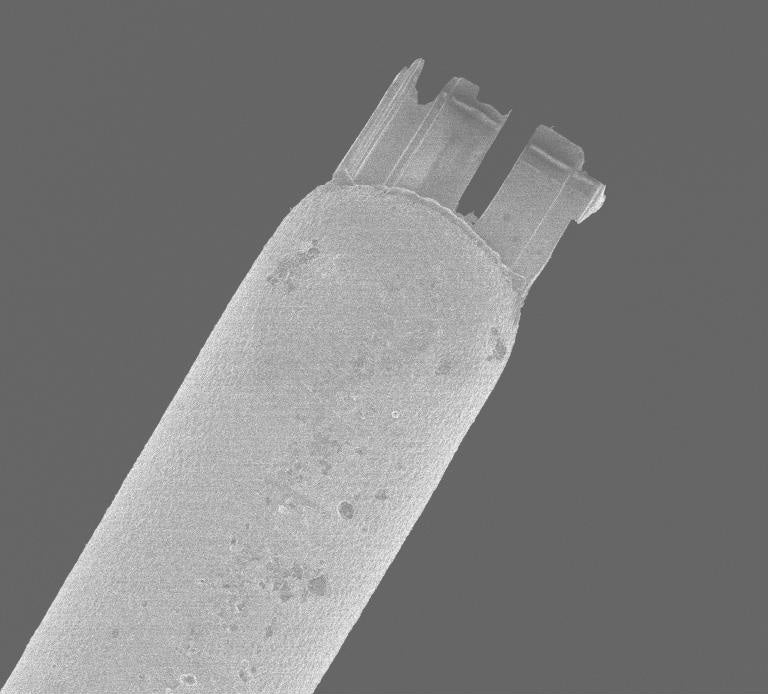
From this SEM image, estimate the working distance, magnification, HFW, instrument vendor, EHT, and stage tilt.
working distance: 4.9 mm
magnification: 0.469 K X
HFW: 243.8 µm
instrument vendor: Zeiss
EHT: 2 kV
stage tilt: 0°